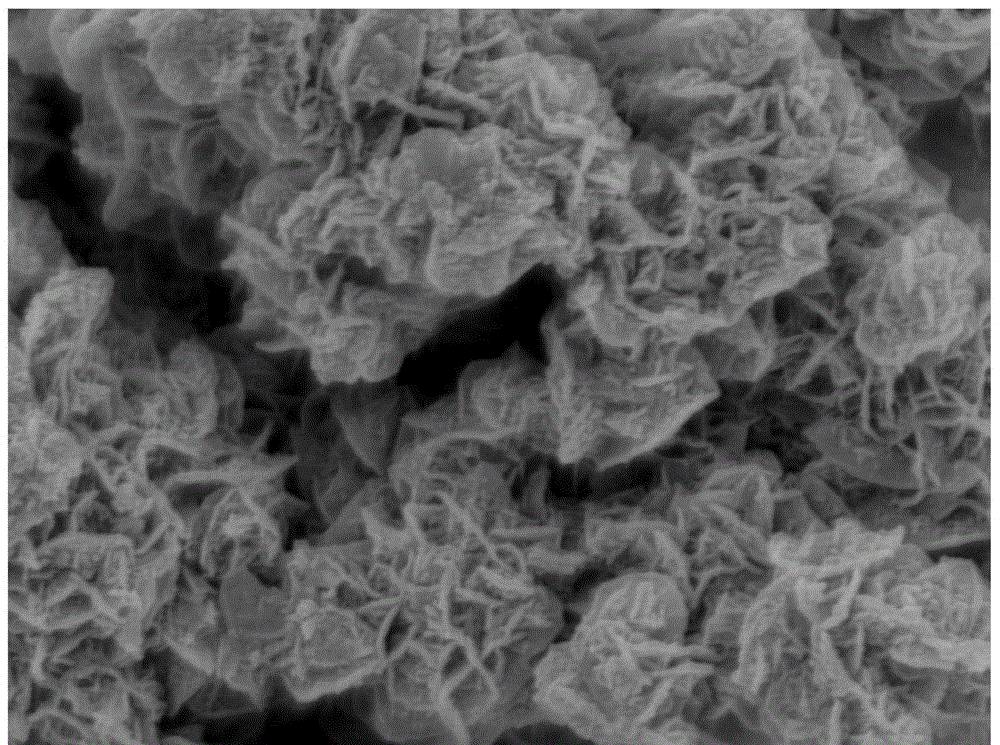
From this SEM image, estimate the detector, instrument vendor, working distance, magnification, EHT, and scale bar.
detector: SEI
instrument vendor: JEOL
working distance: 7.9 mm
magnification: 30 K X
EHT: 5 kV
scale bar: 100 nm